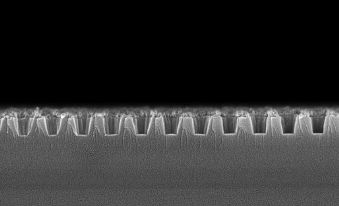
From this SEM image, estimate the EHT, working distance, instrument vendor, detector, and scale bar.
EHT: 5 kV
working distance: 3 mm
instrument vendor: Zeiss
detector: InLens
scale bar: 200 nm